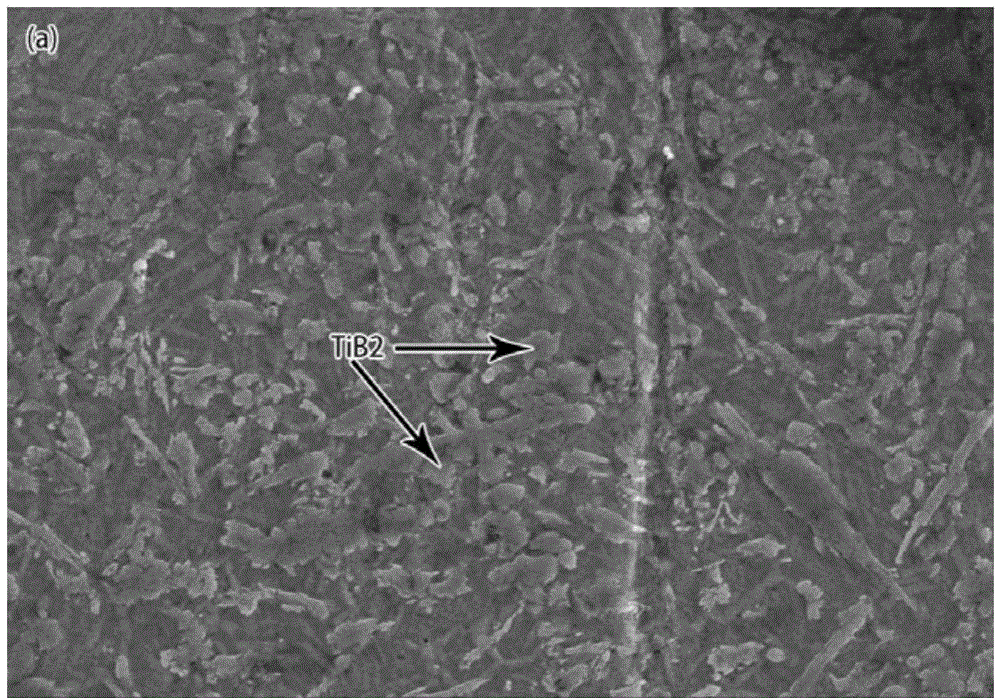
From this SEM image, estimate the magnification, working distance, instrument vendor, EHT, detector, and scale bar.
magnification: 2 K X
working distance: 9.8 mm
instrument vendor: Hitachi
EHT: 15 kV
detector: SE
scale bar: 20000 nm